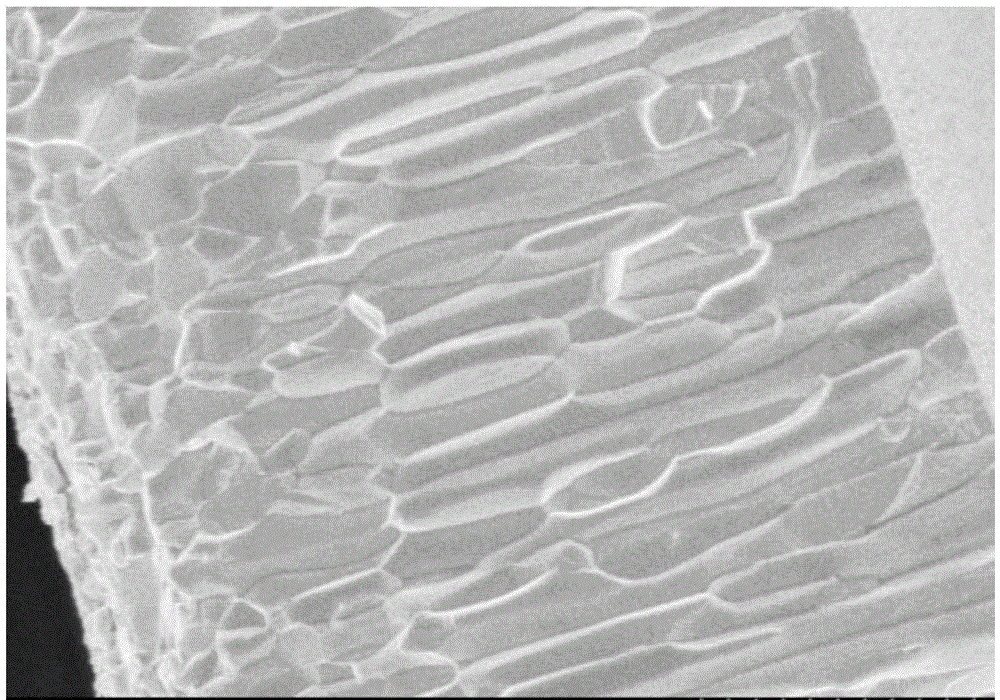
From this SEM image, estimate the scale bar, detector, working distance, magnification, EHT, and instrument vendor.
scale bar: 5000 nm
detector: SE(M)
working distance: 8.4 mm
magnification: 7 K X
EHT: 5 kV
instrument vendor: Hitachi